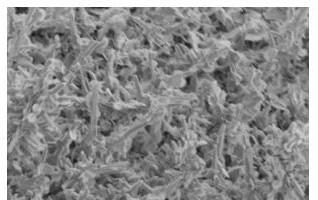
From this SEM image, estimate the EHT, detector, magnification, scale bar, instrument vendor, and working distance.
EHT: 3 kV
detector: SE(M)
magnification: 10 K X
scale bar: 5000 nm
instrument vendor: Hitachi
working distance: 16.5 mm